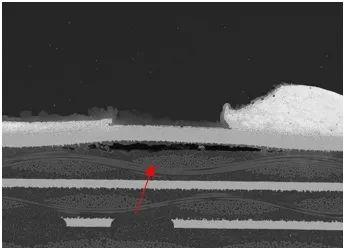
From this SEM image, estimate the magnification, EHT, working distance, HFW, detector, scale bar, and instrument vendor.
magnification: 0.235 K X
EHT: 20 kV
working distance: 12.2 mm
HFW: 1180 µm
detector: BSE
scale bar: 200000 nm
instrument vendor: Tescan